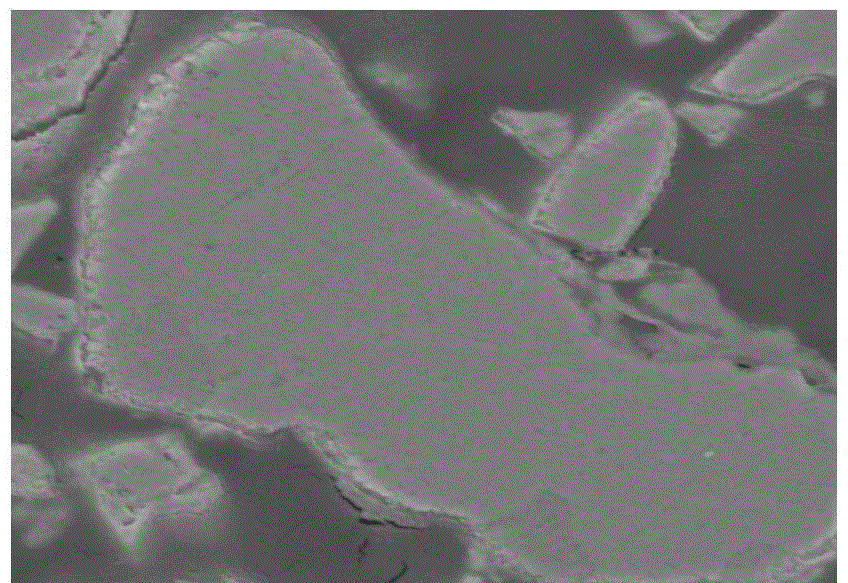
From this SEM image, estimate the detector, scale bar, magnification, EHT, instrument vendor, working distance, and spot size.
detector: ETD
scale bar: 4000 nm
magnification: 20 K X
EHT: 10 kV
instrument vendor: FEI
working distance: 11.5 mm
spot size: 3